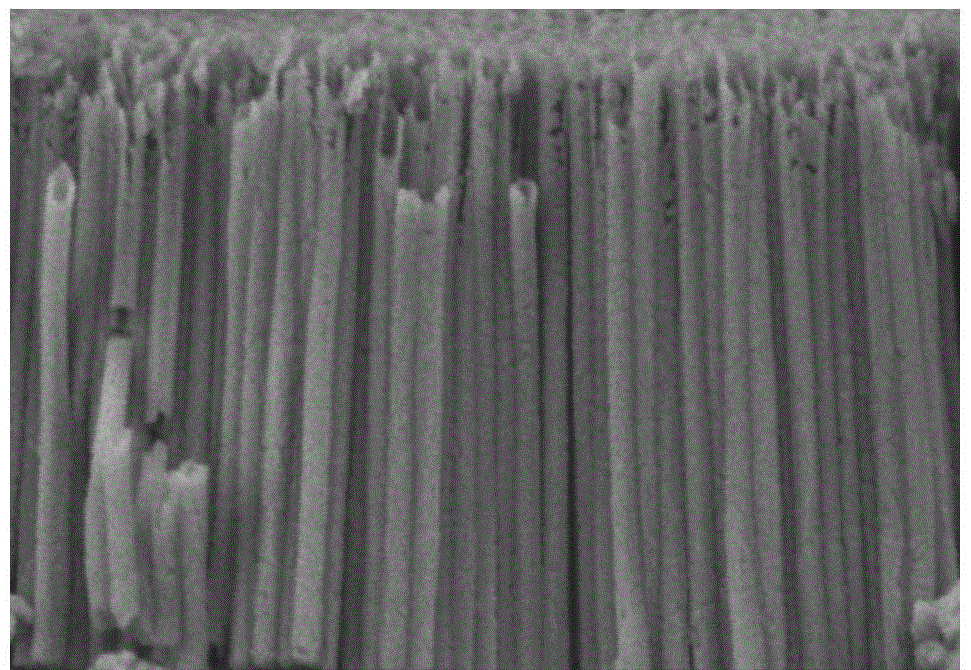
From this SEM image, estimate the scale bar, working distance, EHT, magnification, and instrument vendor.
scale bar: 1000 nm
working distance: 15.5 mm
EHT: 3 kV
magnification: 35 K X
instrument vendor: Hitachi